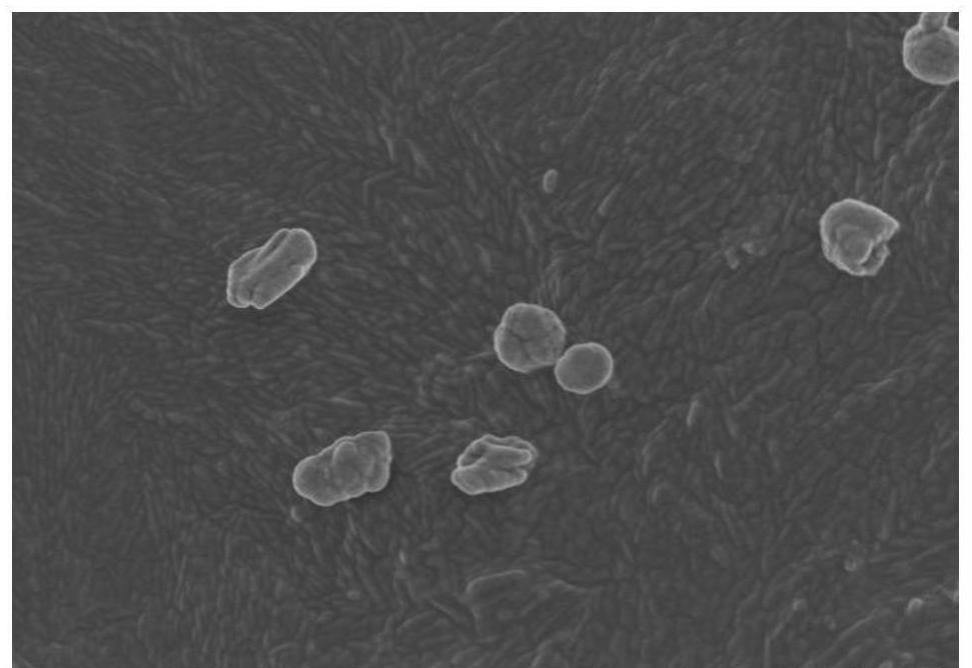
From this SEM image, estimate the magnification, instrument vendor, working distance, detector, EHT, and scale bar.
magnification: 10 K X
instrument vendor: Hitachi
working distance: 5.2 mm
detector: SE(M)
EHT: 3 kV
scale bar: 5000 nm